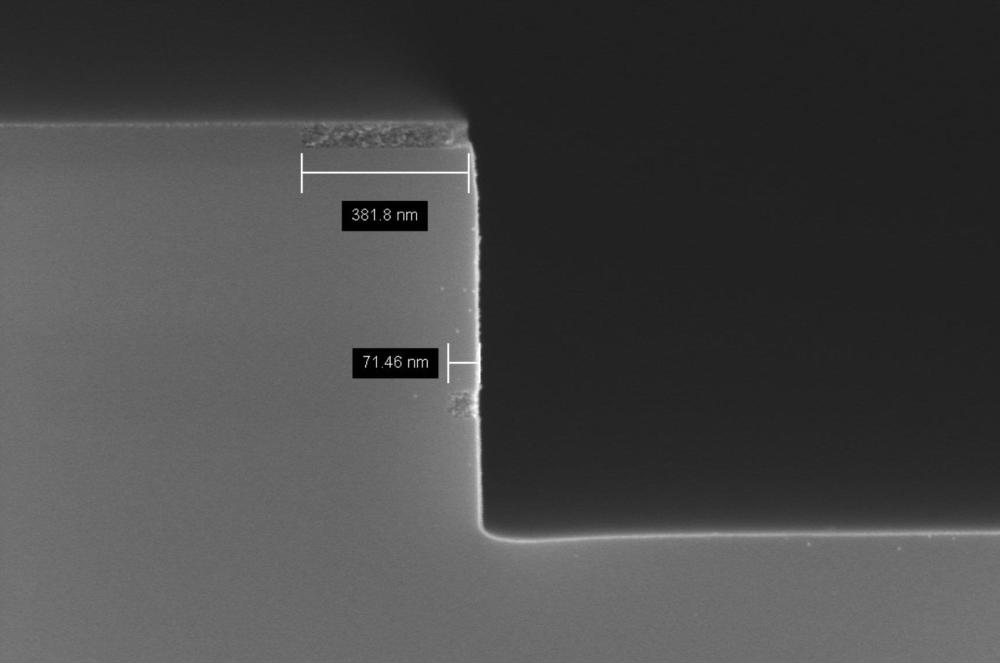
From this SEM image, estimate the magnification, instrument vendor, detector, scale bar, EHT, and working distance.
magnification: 50 K X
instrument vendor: Zeiss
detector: InLens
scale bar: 200 nm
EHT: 5 kV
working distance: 3.6 mm